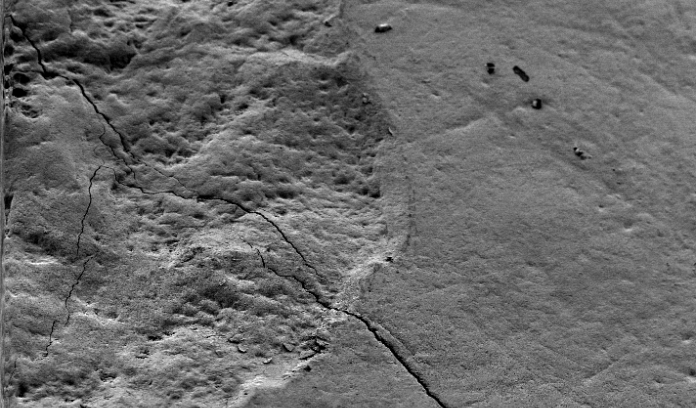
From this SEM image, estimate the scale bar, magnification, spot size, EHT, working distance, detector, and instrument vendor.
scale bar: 50000 nm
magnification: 0.5 K X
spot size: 3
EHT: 5 kV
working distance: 9.9 mm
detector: SE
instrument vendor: FEI